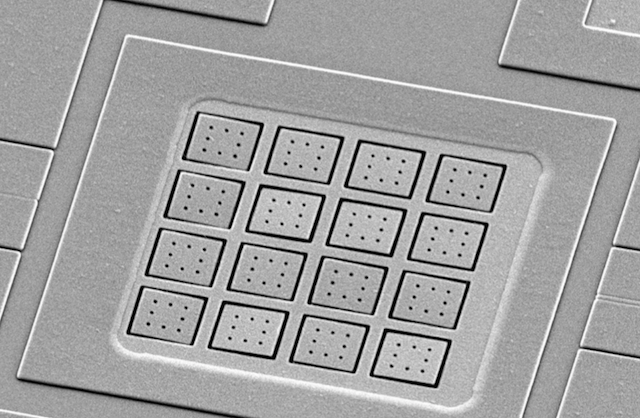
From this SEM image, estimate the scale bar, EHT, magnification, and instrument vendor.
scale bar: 20000 nm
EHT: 10 kV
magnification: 0.7 K X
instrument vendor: JEOL